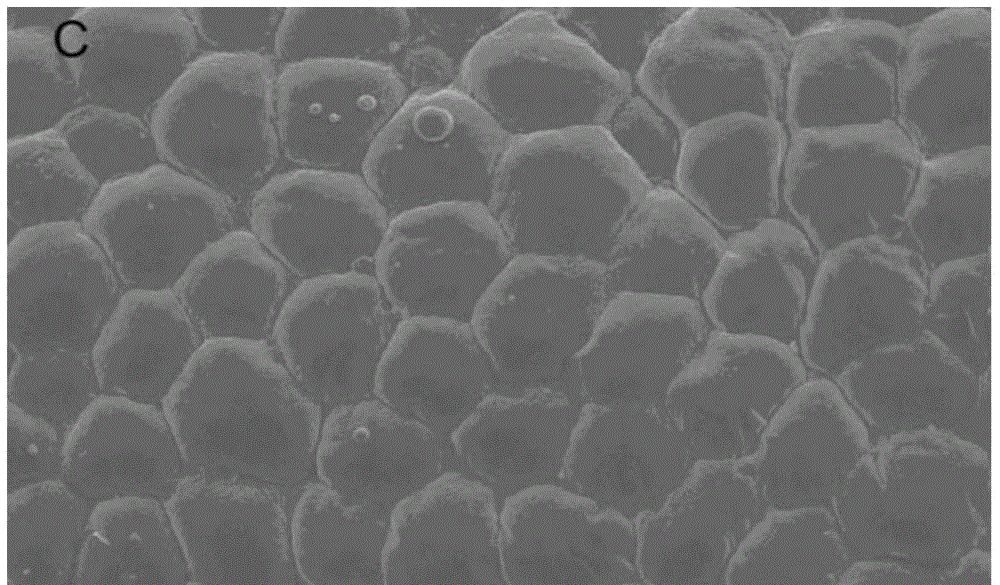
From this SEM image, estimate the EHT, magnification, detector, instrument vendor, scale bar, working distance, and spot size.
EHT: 20 kV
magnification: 0.2 K X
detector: SE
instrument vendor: FEI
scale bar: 100000 nm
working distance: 18.7 mm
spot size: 3